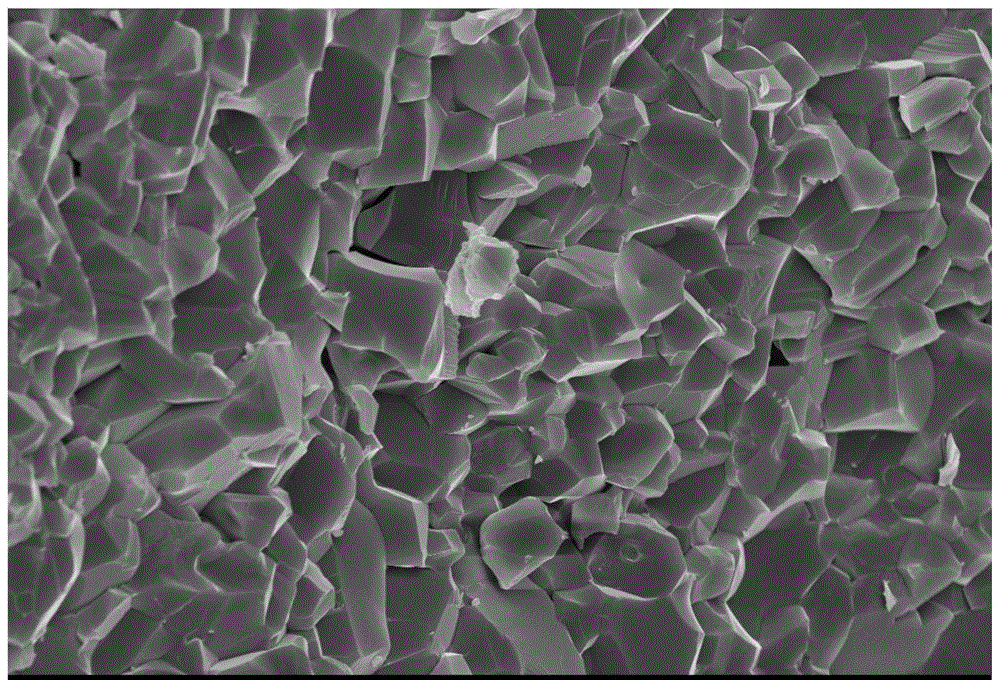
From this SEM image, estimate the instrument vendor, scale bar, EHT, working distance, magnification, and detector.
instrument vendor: Zeiss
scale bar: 2000 nm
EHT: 15 kV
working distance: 7.1 mm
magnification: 3 K X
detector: InLens